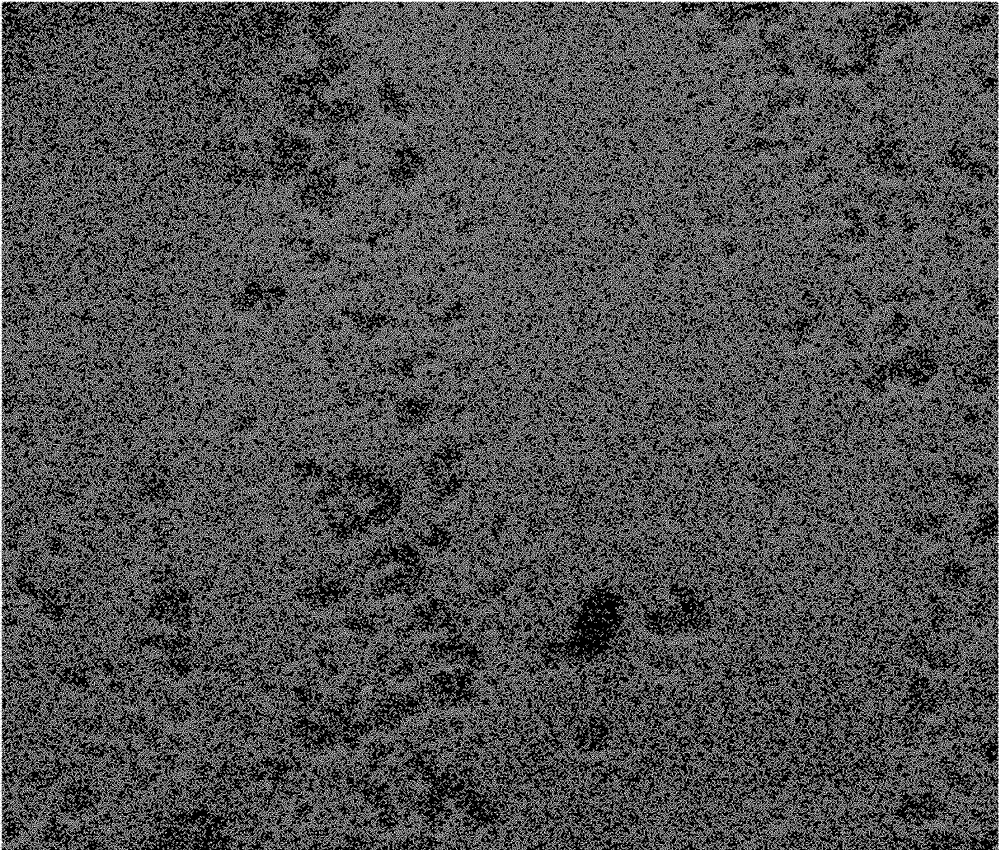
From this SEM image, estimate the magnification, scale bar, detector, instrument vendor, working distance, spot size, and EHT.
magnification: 100 K X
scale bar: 500 nm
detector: ETD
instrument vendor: FEI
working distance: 6.4 mm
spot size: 3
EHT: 15 kV